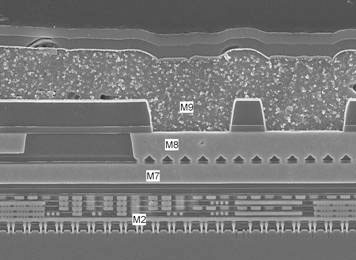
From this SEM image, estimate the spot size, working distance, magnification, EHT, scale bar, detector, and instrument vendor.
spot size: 3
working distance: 5 mm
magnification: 8 K X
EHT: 10 kV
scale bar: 2000 nm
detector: TLD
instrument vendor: FEI